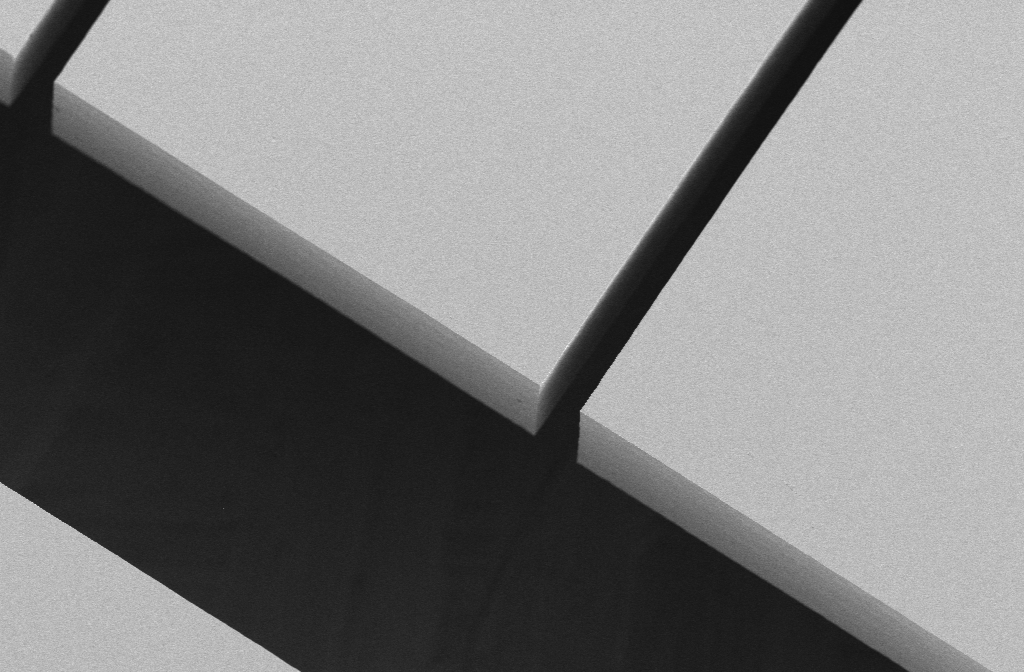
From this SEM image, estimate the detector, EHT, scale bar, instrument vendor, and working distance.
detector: SE2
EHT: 2 kV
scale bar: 20000 nm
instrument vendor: Zeiss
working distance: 5 mm